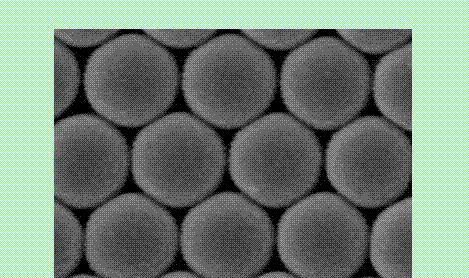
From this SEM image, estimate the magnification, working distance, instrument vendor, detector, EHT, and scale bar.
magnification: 120 K X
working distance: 6.9 mm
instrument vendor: Hitachi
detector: SE(M)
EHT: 15 kV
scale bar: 400 nm